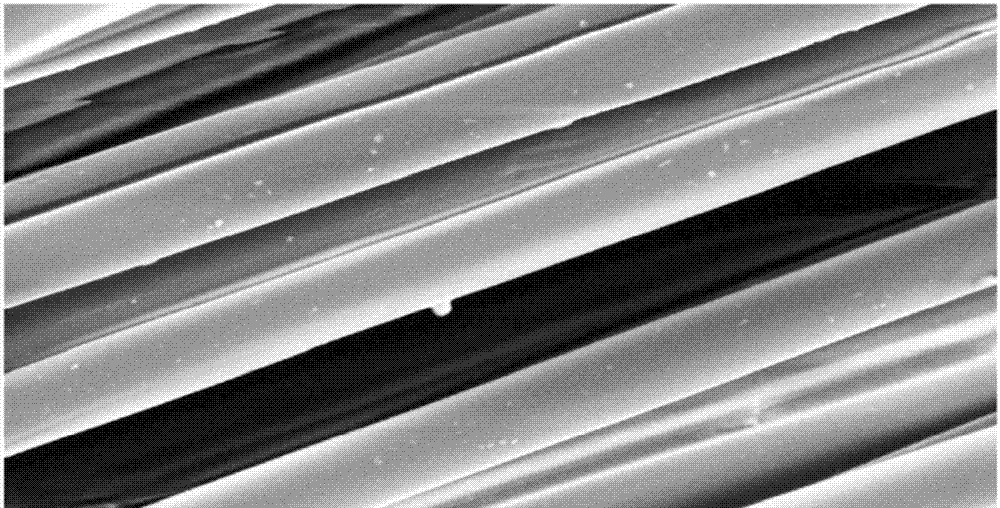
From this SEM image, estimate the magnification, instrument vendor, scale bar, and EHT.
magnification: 1 K X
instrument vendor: other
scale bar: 20000 nm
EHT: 10 kV